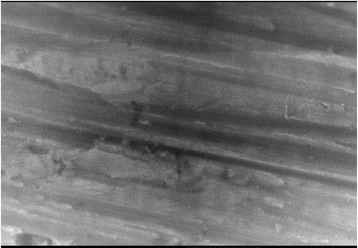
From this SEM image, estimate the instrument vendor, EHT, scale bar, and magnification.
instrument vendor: JEOL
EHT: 20 kV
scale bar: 5000 nm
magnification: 5 K X